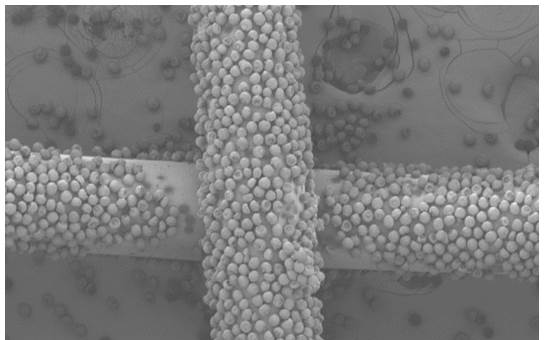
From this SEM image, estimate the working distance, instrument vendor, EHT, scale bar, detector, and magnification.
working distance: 12 mm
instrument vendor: Hitachi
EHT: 1 kV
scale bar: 500000 nm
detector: SE(M)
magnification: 0.1 K X